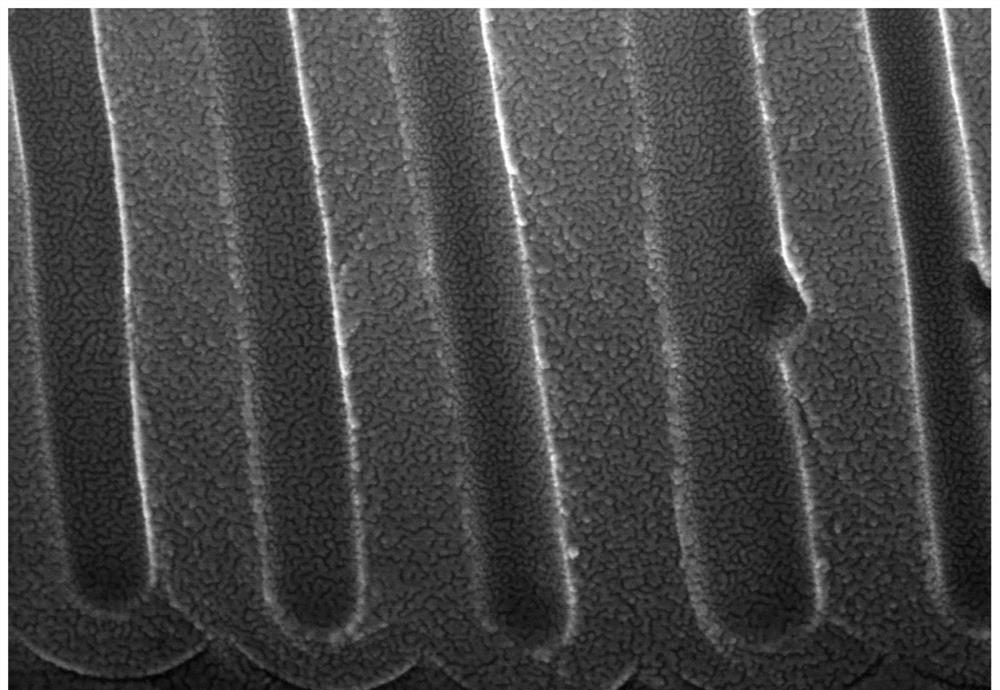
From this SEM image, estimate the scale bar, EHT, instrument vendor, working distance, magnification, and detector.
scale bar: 500 nm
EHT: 15 kV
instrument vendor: Hitachi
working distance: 8.3 mm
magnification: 100 K X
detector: SE(UL)